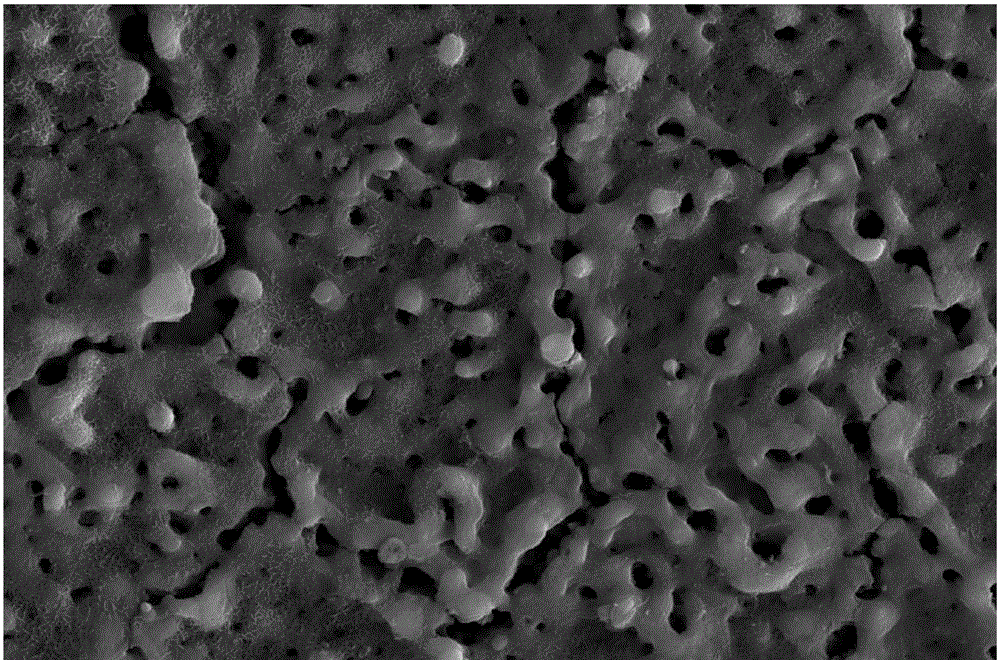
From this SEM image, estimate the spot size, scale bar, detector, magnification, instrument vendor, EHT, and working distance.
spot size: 4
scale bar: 5000 nm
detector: ETD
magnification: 20 K X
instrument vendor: FEI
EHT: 15 kV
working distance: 7.3 mm